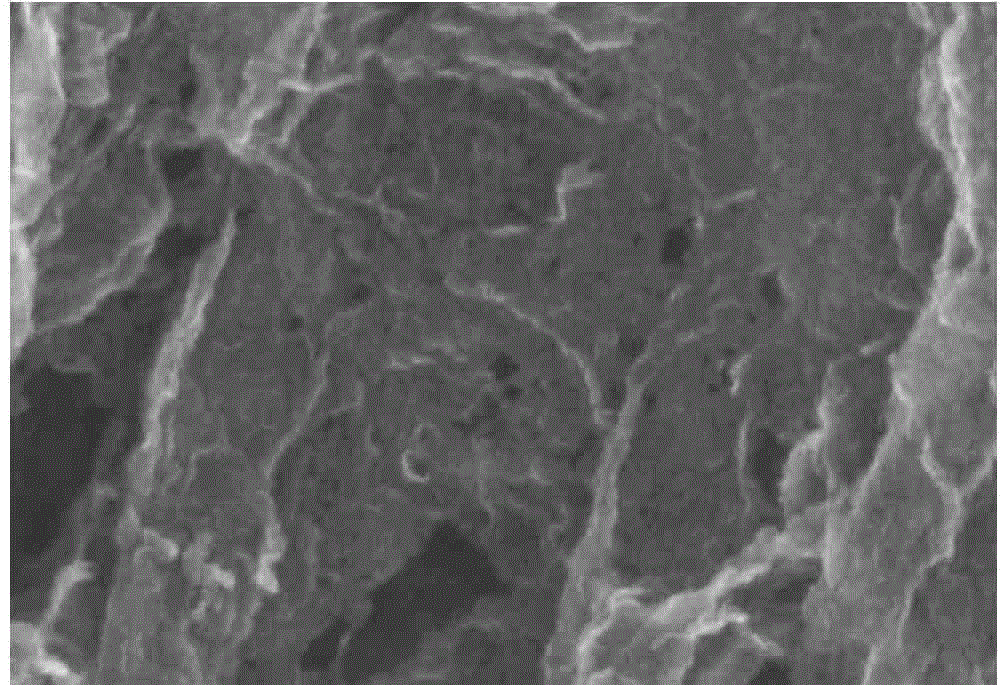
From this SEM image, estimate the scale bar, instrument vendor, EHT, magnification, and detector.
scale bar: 500 nm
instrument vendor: Hitachi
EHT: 6 kV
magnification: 110 K X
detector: SE(U)